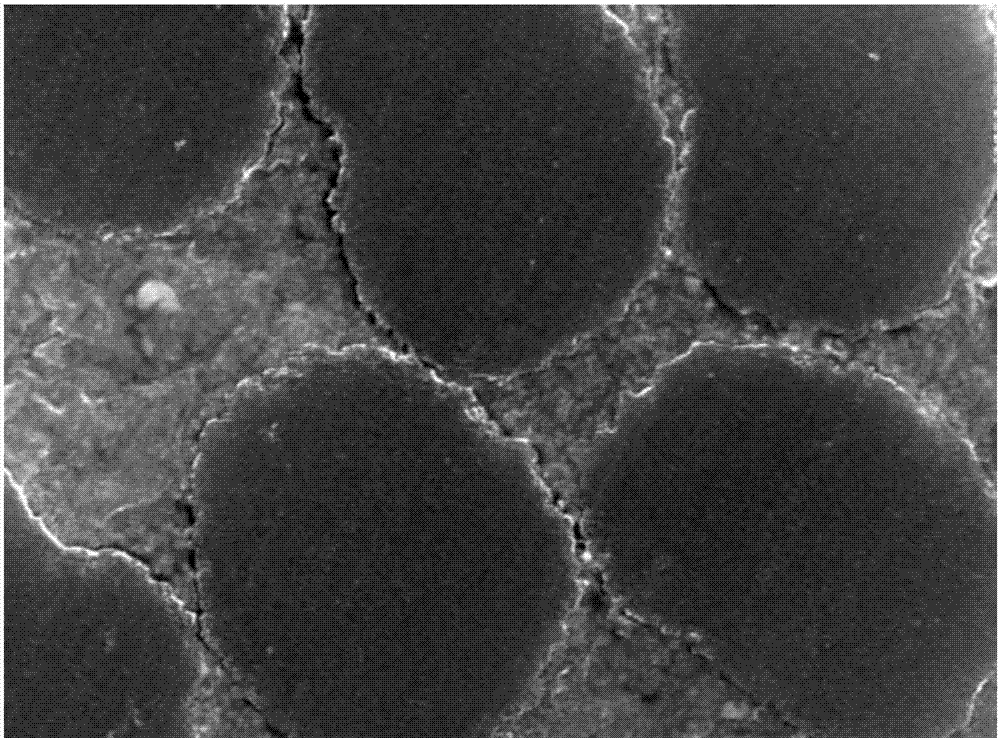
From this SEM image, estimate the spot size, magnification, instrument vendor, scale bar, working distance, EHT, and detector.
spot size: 5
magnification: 10 K X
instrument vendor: FEI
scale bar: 2000 nm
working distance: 26.6 mm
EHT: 20 kV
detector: SE